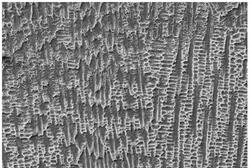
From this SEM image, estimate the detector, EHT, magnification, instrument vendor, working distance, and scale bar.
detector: SE1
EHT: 20 kV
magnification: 0.5 K X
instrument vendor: Zeiss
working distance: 10.5 mm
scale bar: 10000 nm